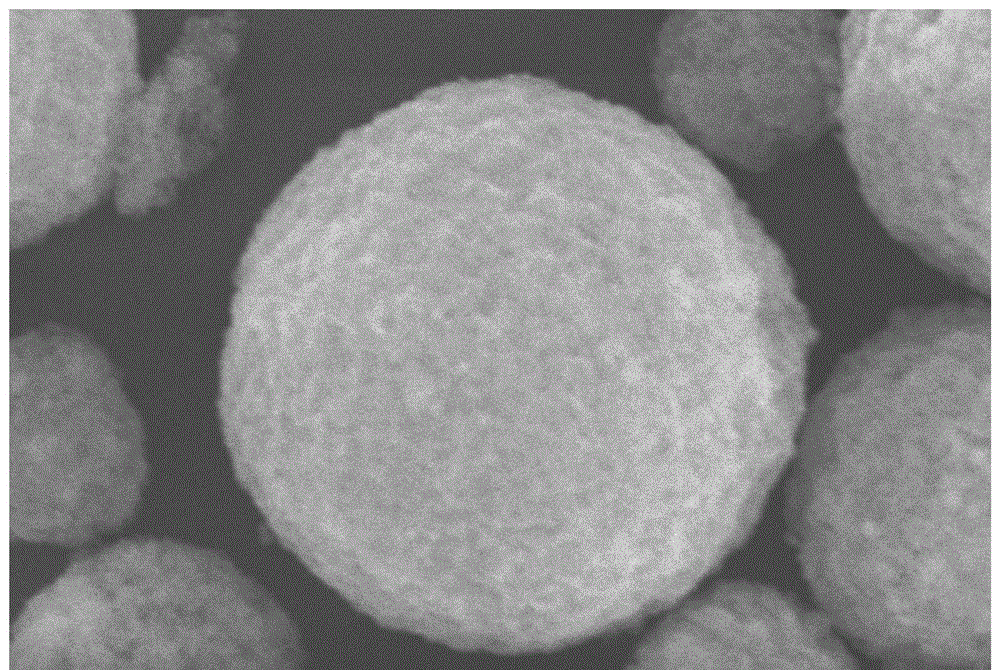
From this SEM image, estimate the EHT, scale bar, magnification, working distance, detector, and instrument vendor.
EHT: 20 kV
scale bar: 2000 nm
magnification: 7 K X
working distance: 10 mm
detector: SED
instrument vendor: JEOL